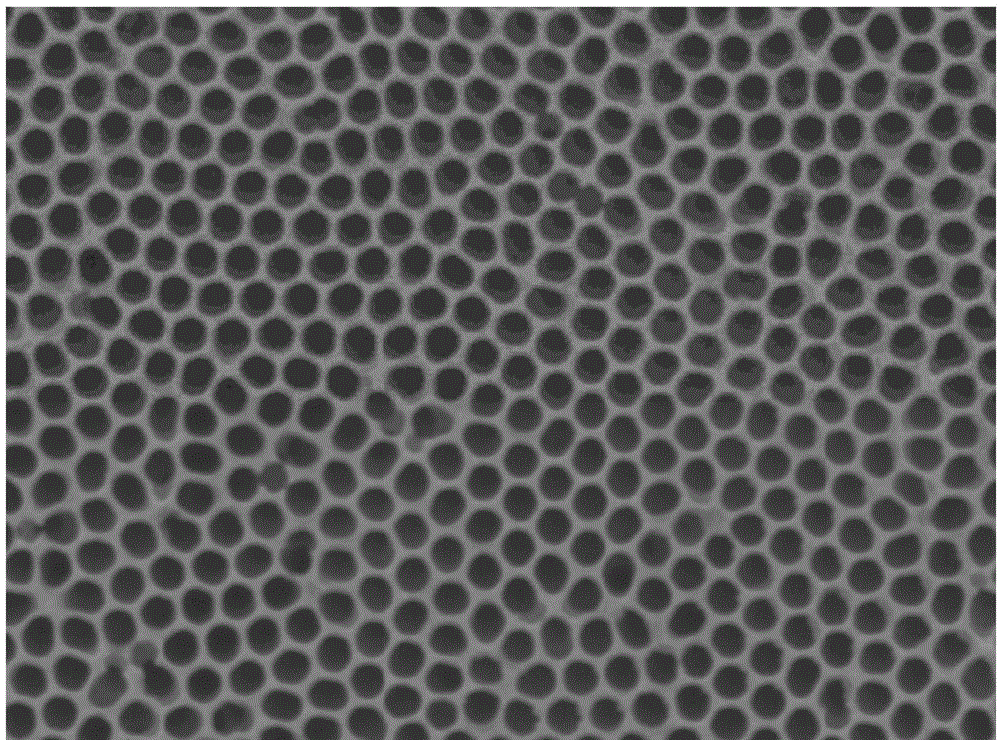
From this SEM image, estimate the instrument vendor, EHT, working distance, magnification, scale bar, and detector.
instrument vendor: JEOL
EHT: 15 kV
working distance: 12.3 mm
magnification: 50 K X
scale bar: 100 nm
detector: SEI